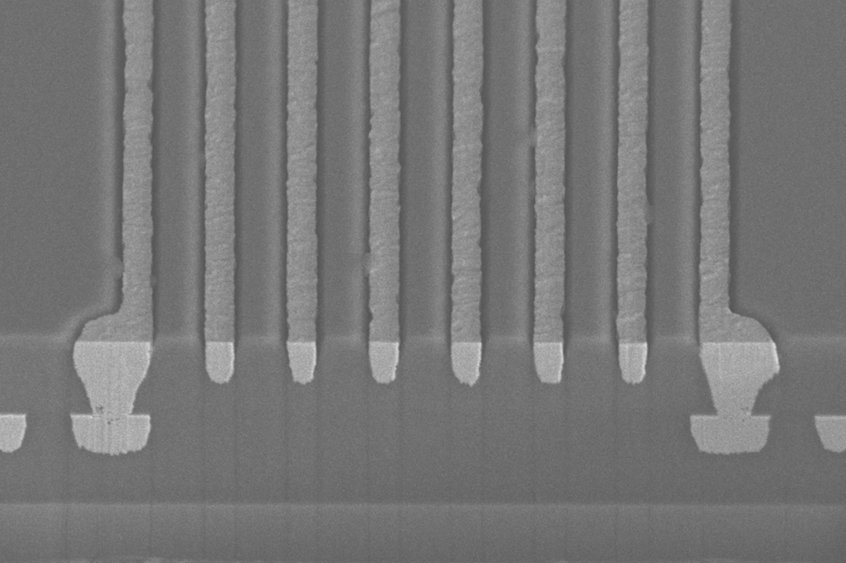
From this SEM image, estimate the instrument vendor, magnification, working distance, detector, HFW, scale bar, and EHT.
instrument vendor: FEI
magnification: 2 K X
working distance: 4 mm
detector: TLD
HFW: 104 µm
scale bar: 30000 nm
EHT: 15 kV